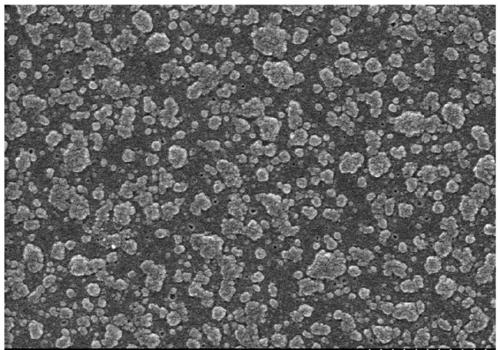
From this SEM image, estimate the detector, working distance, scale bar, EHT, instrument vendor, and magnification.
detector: SE(U)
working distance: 5.1 mm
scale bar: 1000 nm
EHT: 3 kV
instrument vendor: Hitachi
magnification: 30 K X